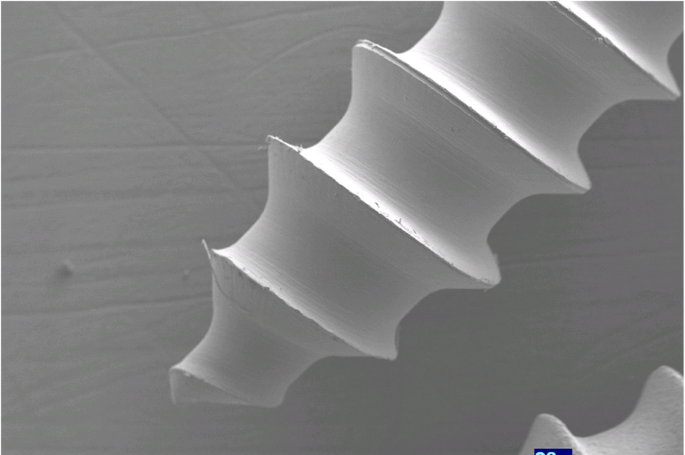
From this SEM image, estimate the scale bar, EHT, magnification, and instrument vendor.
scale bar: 1000000 nm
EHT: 23 kV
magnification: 0.0274 K X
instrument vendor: other